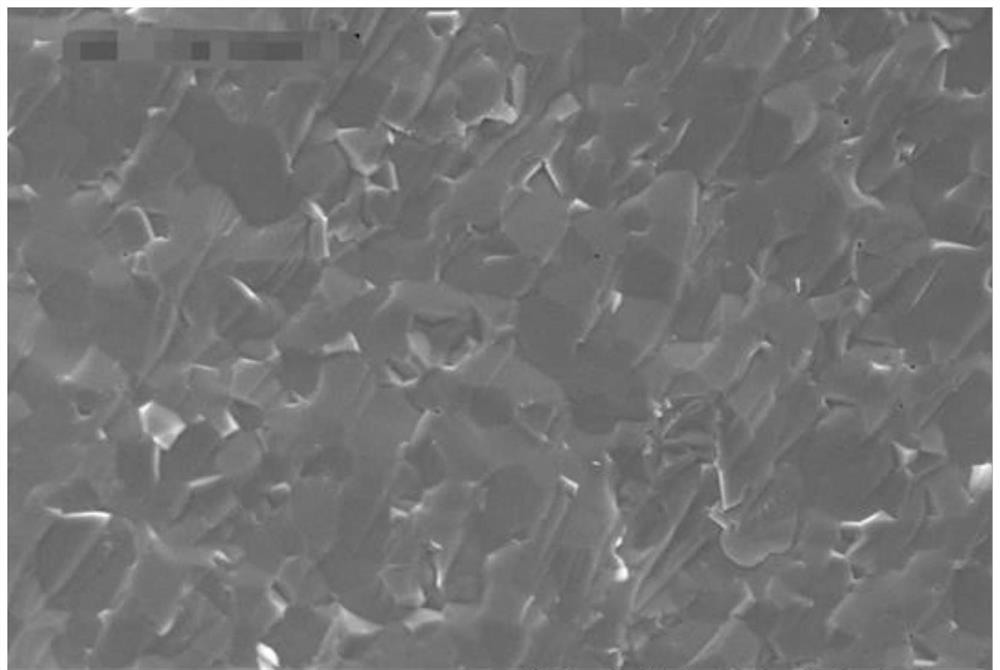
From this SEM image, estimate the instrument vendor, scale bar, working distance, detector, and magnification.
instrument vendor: Hitachi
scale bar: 50000 nm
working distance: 14.7 mm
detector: SE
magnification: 1 K X